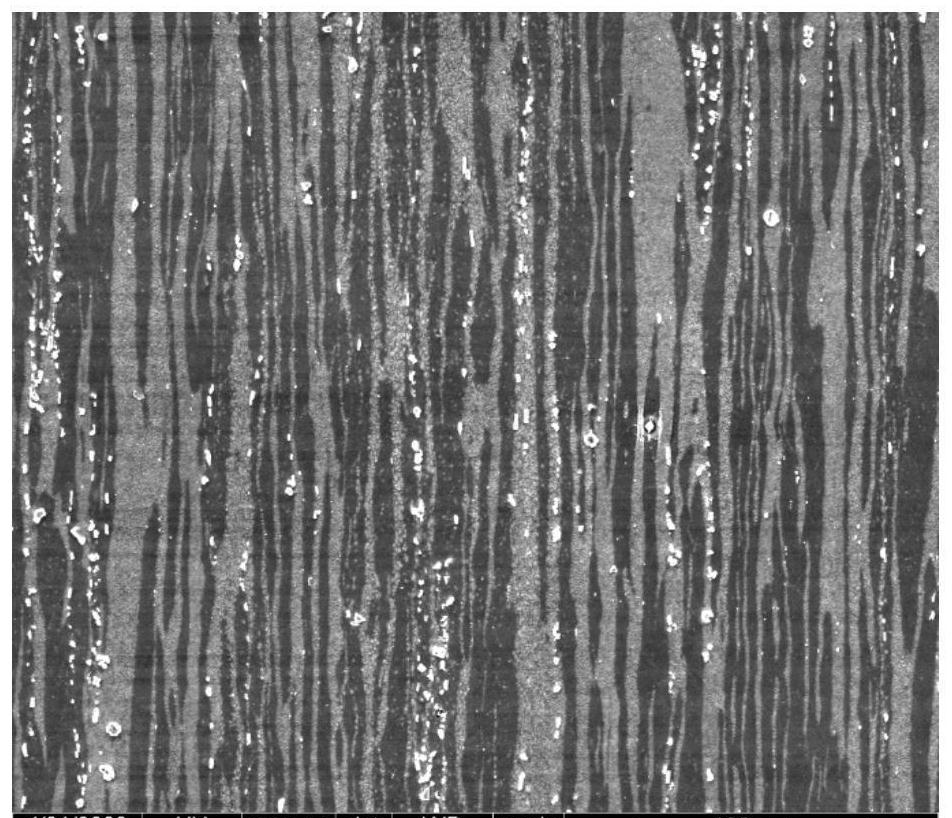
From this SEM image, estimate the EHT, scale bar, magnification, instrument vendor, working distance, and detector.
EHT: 20 kV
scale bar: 100000 nm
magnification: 0.5 K X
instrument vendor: FEI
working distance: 11.5 mm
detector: ETD